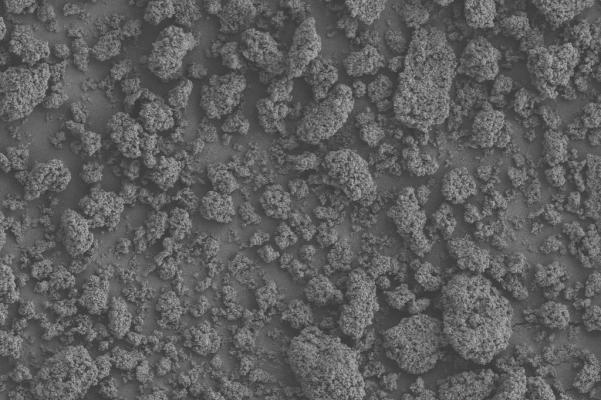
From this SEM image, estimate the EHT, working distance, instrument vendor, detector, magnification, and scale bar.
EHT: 3 kV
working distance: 8.2 mm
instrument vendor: Zeiss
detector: SE2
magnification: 1 K X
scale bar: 10000 nm